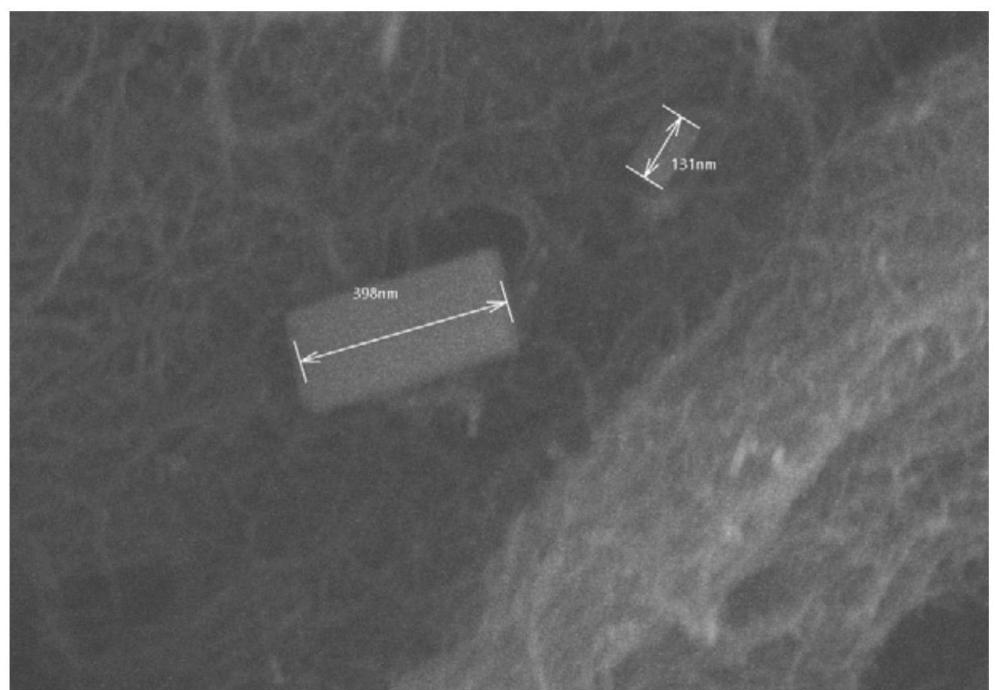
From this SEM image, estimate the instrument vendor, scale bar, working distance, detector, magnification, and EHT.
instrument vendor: Hitachi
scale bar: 500 nm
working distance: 9.8 mm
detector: SE(UL)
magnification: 70 K X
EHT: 10 kV